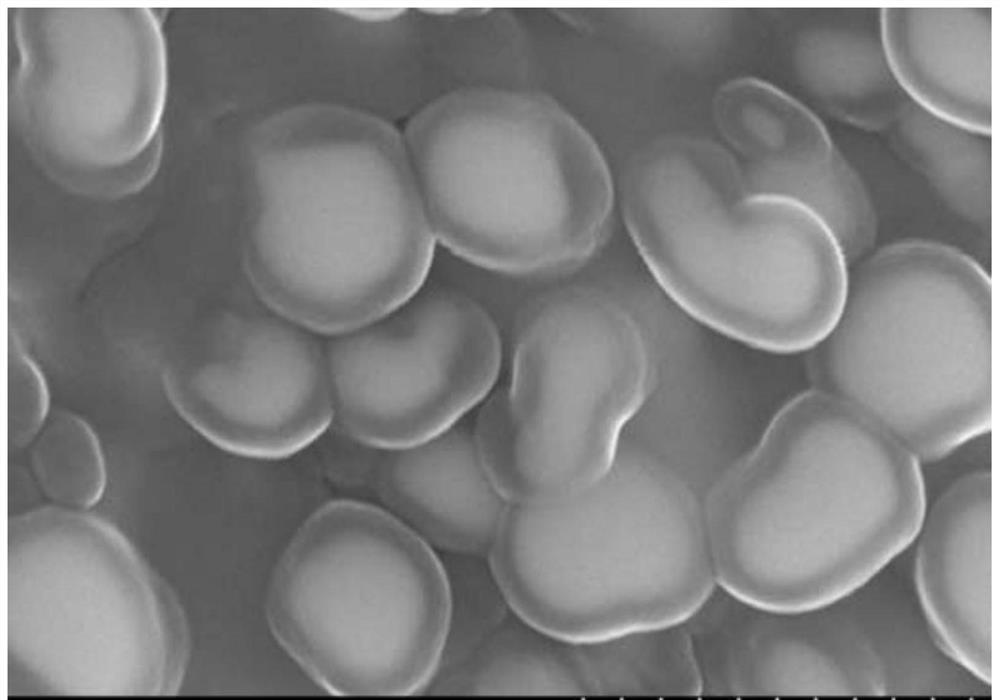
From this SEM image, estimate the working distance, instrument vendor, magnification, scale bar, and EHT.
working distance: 4.9 mm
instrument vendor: Hitachi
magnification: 100 K X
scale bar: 100 nm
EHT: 10 kV